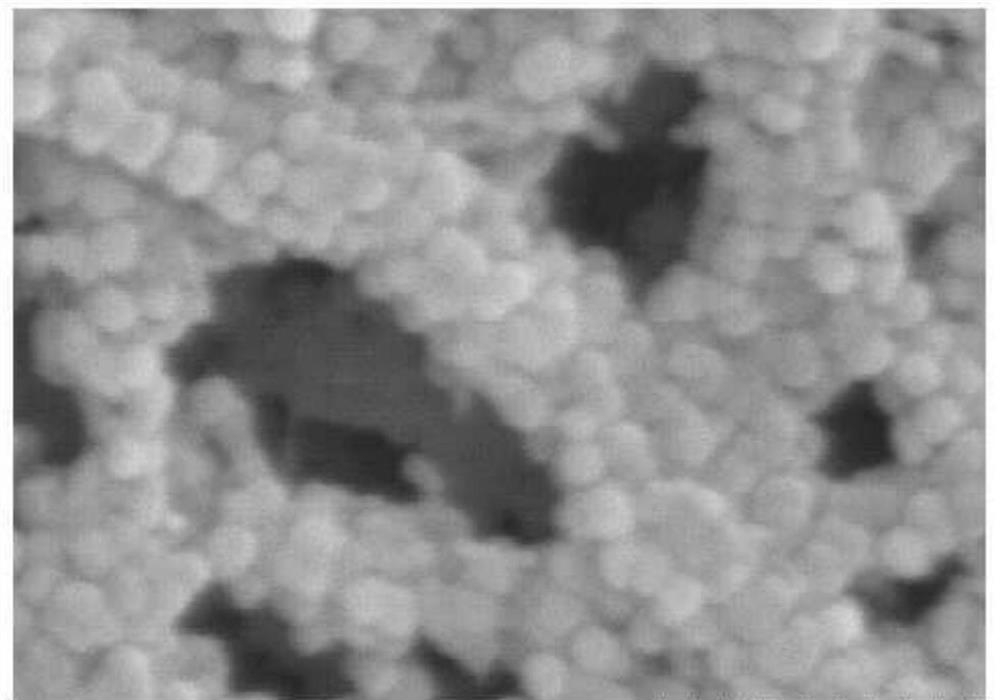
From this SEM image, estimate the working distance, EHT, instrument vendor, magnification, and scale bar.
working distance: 13.5 mm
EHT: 5 kV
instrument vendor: Hitachi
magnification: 200 K X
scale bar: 200 nm